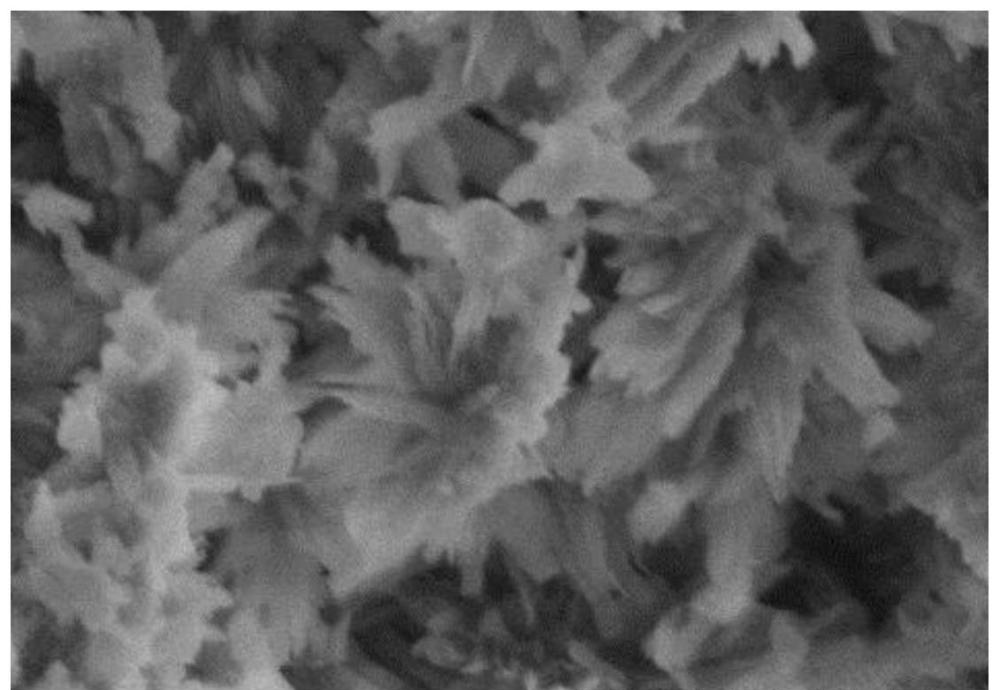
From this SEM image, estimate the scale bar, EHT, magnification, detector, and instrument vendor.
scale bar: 1000 nm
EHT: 3 kV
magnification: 50 K X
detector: SE(U)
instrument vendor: Hitachi